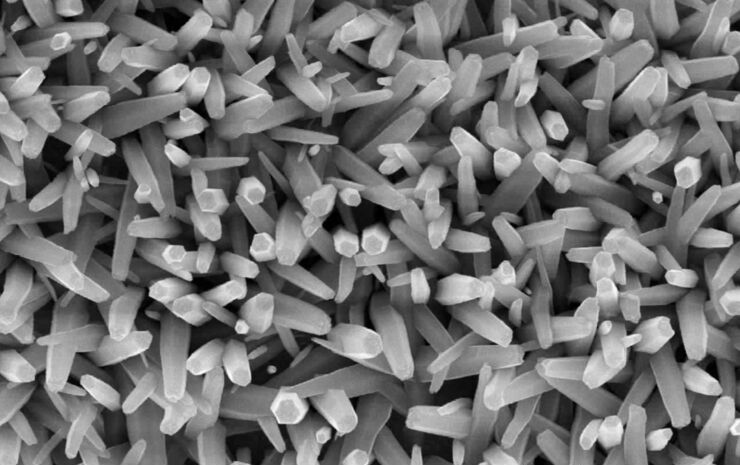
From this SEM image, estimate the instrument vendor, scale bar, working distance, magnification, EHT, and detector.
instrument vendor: JEOL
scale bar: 1000 nm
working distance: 9.6 mm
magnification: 8 K X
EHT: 15 kV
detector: SEI